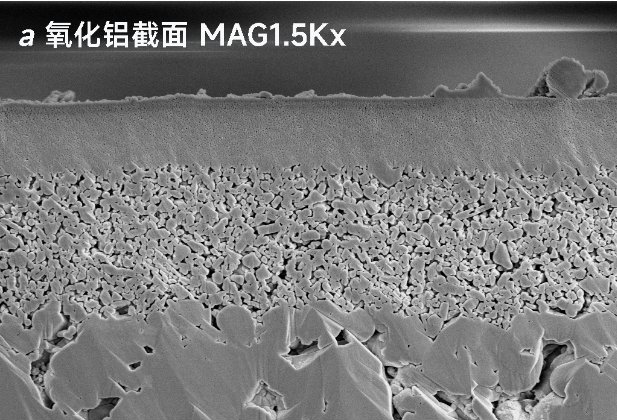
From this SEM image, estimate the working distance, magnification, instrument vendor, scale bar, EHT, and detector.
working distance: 11.121 mm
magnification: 1.5 K X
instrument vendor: other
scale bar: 20000 nm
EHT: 10 kV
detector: SE